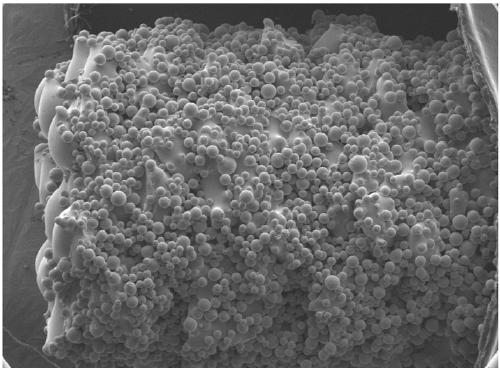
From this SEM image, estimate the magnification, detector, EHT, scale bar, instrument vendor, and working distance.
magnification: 0.035 K X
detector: SEI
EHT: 10 kV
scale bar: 100000 nm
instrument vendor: JEOL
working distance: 12 mm